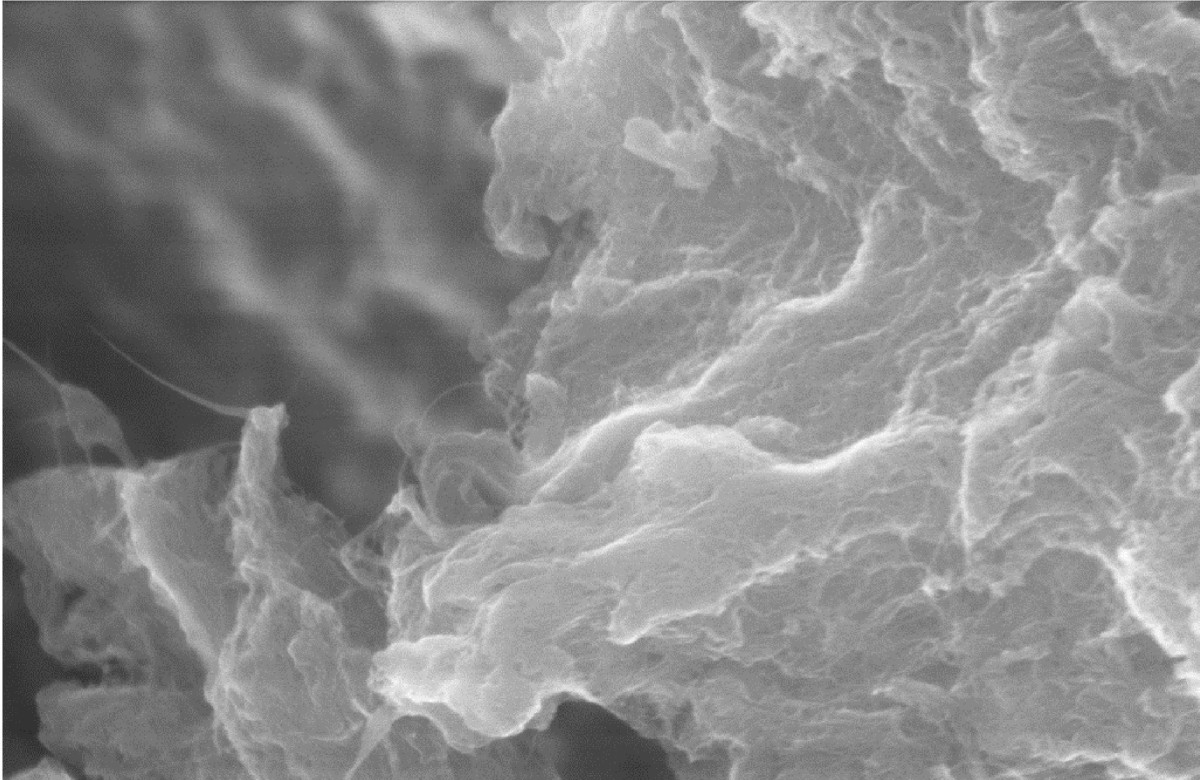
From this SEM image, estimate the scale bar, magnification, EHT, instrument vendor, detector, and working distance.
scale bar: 200 nm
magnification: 37.35 K X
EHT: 10 kV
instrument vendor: Zeiss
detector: InLens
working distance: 6 mm